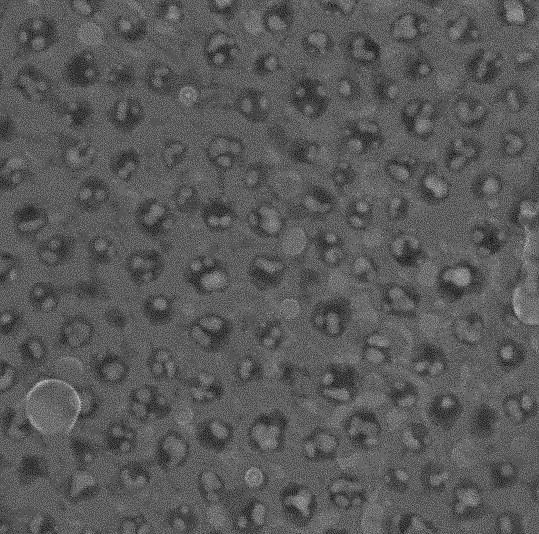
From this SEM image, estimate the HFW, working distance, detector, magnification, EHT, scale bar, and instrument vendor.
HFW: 0.963 µm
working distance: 2.819 mm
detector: InBeam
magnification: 300 K X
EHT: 15 kV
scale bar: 200 nm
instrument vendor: Tescan